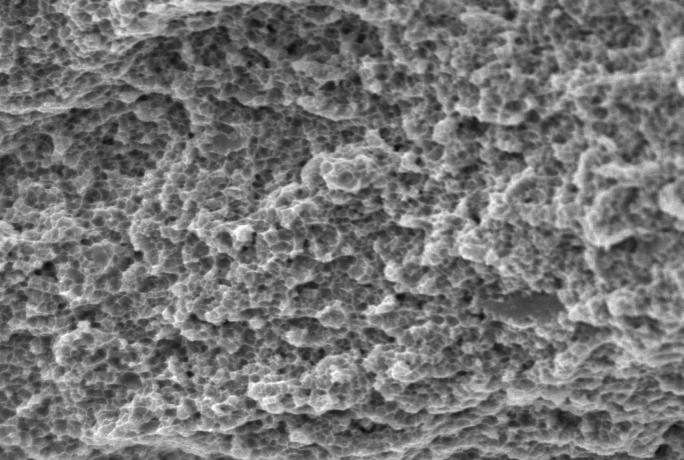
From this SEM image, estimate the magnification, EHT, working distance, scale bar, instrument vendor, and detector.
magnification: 3 K X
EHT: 20 kV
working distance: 15.5 mm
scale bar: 10000 nm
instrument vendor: Hitachi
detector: SE